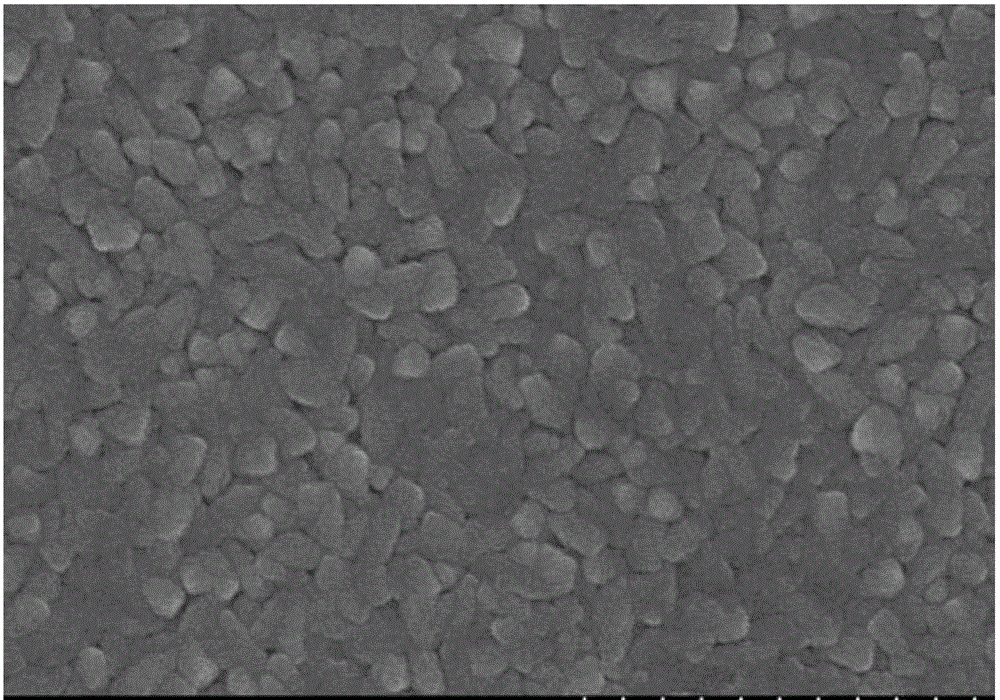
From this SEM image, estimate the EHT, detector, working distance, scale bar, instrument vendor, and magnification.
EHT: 10 kV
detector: SE(M)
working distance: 7.7 mm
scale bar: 1000 nm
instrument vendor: Hitachi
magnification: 50 K X